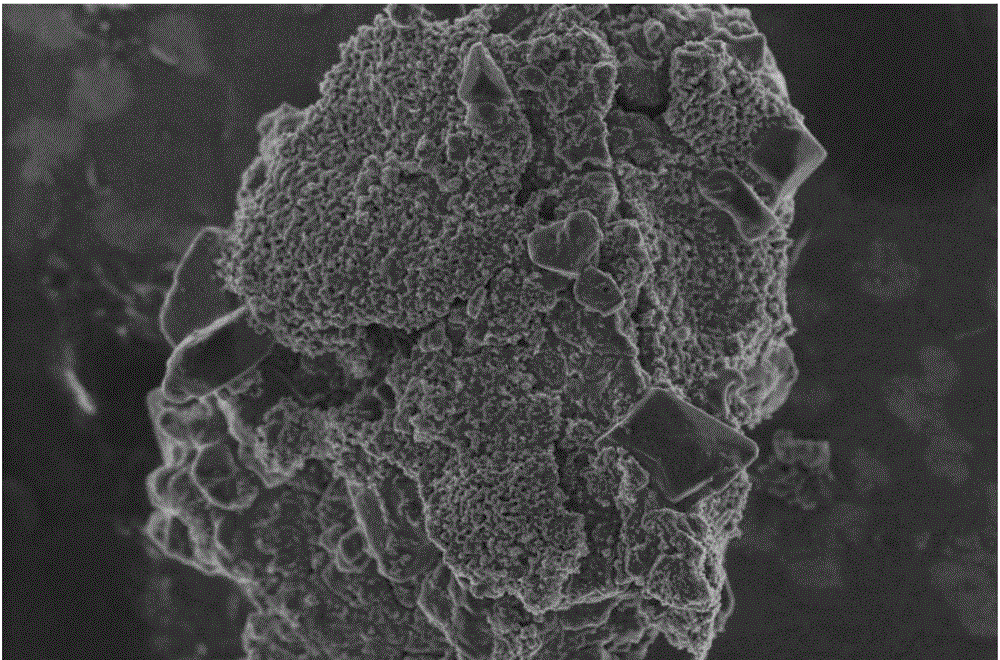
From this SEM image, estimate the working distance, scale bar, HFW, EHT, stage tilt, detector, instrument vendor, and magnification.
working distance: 4 mm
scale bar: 1000 nm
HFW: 8.29 µm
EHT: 2 kV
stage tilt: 0°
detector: TLD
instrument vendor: FEI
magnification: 50 K X